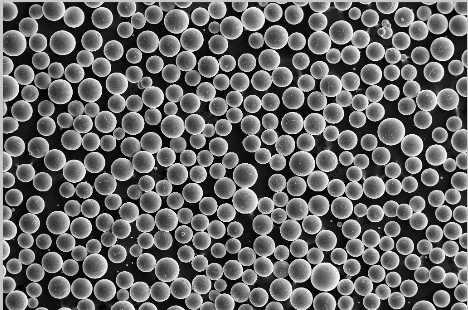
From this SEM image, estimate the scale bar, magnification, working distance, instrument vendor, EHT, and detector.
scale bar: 100000 nm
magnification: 0.05 K X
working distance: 18 mm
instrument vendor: Zeiss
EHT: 20 kV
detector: SE1